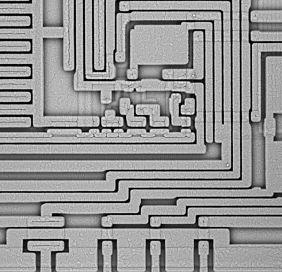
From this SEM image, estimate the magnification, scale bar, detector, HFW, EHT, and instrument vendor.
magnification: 1.5 K X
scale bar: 50000 nm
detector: BSD full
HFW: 170 µm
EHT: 10 kV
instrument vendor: Thermo